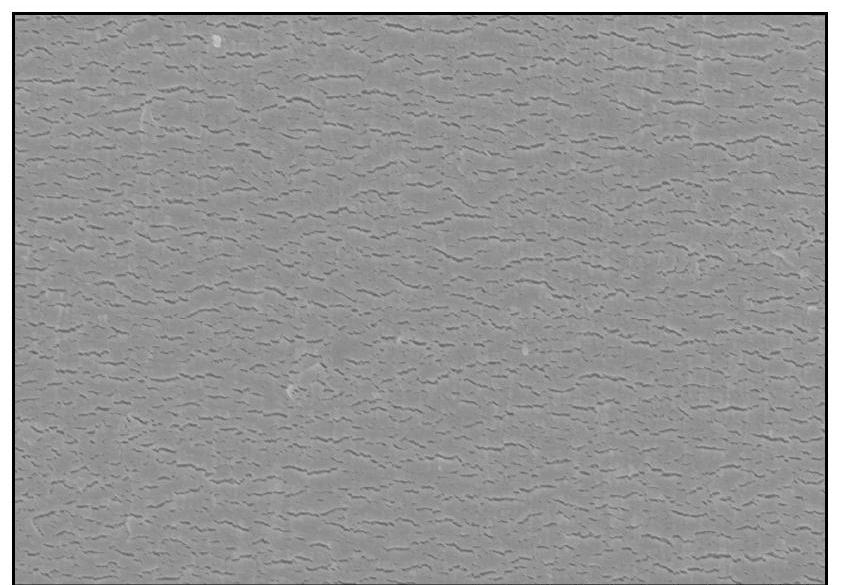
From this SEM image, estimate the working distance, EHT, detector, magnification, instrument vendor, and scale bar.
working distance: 11.4 mm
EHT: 3 kV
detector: SE(U)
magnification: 13 K X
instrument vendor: Hitachi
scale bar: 4000 nm